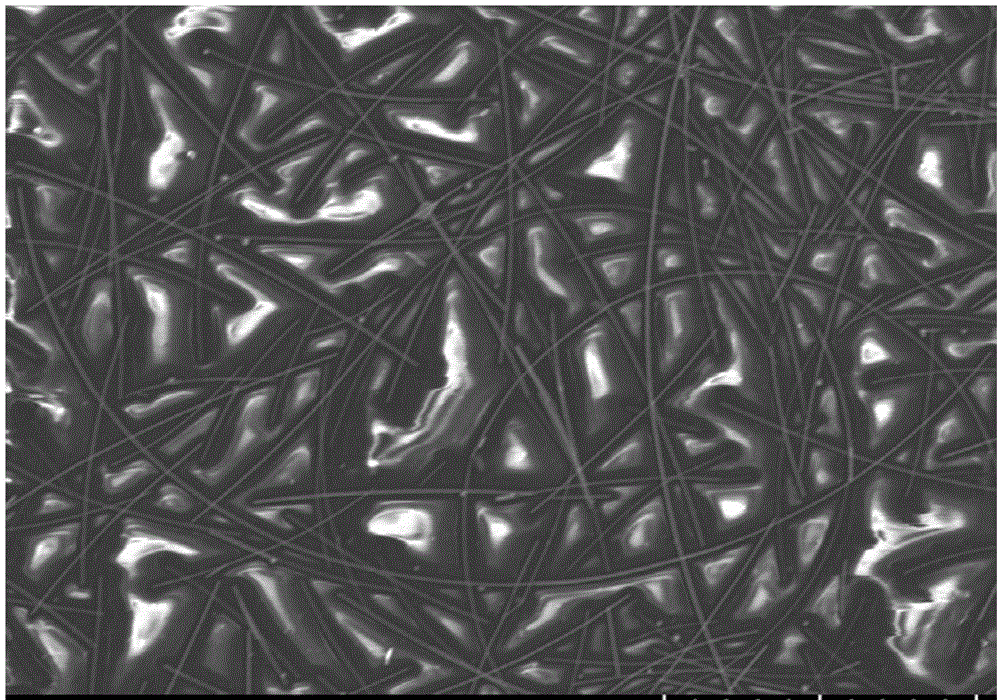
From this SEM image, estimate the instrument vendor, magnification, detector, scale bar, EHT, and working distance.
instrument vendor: Hitachi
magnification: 2 K X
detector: SE(TUL)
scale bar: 20000 nm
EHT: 5 kV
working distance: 11.2 mm